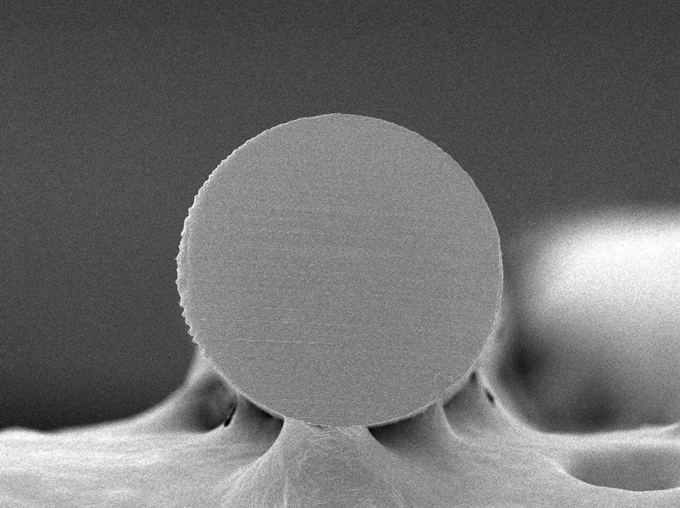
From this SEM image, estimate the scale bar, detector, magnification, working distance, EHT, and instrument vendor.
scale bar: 100000 nm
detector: SEI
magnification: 0.15 K X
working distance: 12.3 mm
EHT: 5 kV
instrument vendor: JEOL